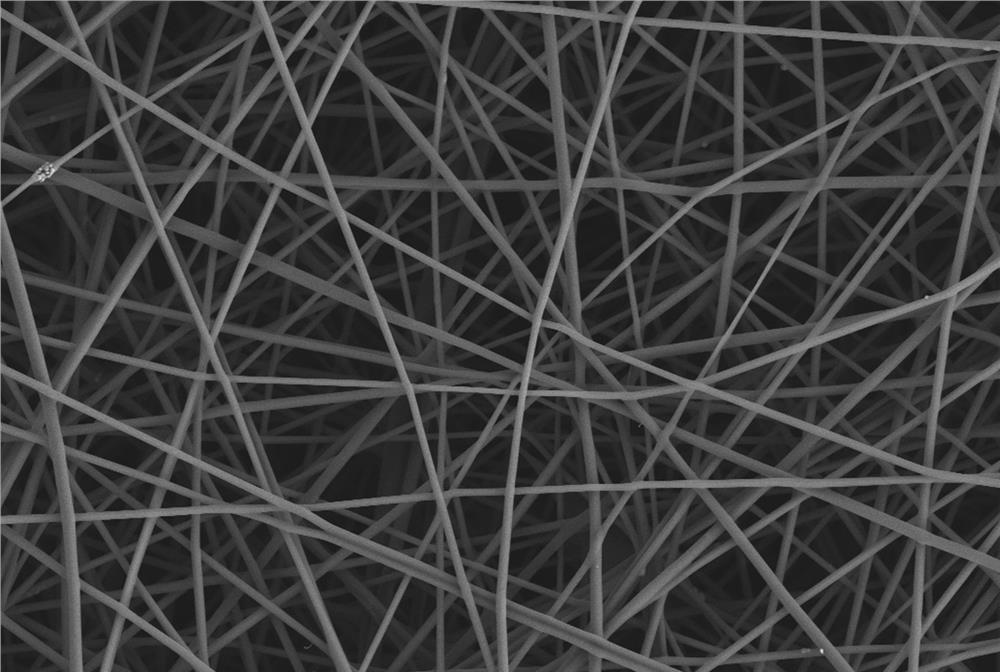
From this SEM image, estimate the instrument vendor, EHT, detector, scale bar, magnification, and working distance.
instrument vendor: Zeiss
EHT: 3 kV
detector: SE2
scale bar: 1000 nm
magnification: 5 K X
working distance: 5.4 mm